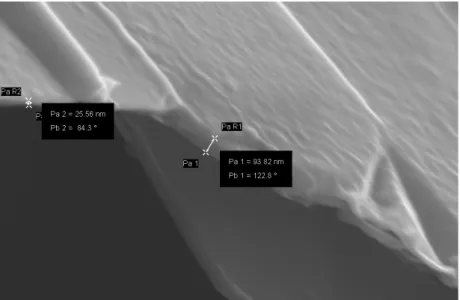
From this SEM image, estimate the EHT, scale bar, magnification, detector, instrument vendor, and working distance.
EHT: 5 kV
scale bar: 200 nm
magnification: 115.65 K X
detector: InLens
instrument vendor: Zeiss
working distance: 3.8 mm